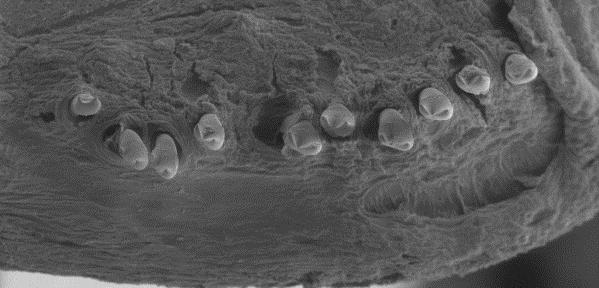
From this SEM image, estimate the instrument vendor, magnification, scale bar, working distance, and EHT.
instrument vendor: other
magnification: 0.105 K X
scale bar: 200000 nm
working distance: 6 mm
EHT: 15 kV